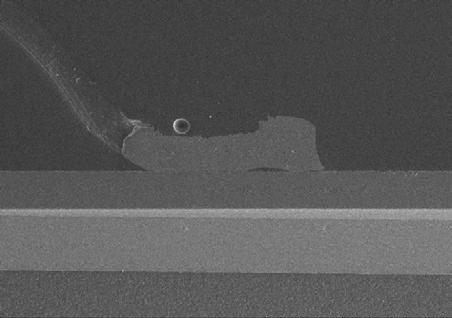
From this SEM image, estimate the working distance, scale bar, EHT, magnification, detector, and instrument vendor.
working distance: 14.6 mm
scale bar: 1000000 nm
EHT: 15 kV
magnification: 0.05 K X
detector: SE(M)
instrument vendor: Hitachi